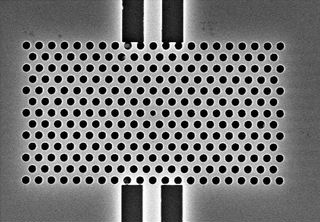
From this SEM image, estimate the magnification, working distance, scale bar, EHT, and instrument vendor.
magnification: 10 K X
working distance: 4 mm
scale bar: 2000 nm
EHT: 10 kV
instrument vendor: other